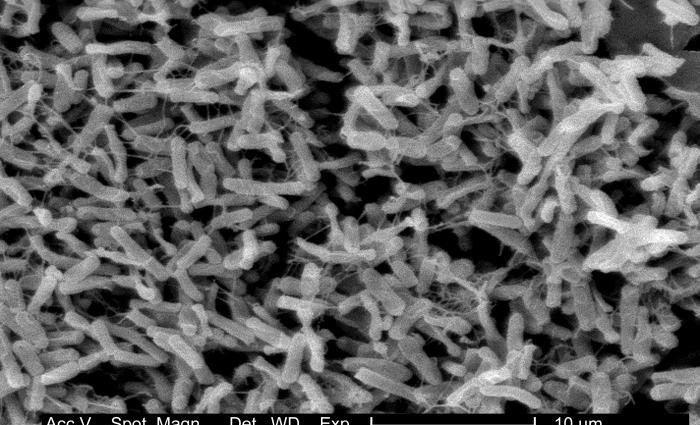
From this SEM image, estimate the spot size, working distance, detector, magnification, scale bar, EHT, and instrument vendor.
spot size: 3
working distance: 33.5 mm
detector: SE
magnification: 2.905 K X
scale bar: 10000 nm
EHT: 10 kV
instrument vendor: FEI